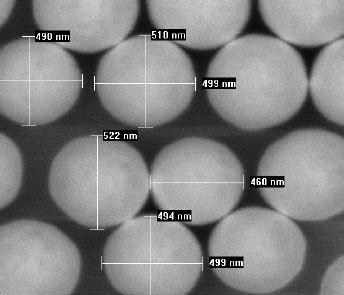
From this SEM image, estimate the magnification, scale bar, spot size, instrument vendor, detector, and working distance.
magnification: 50 K X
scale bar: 500 nm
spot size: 3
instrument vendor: FEI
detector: TLD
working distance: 5 mm